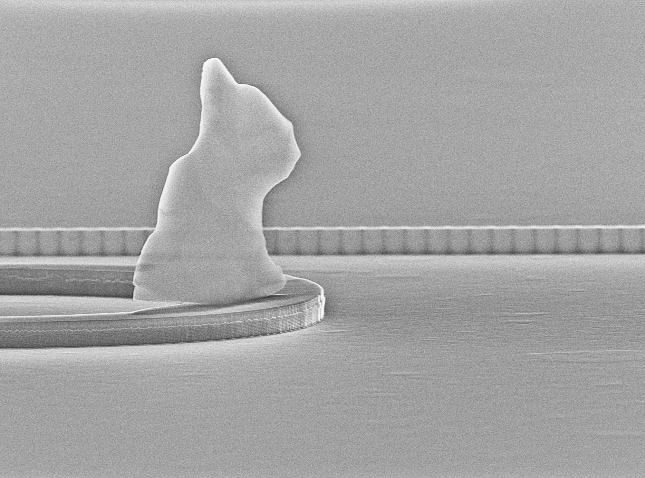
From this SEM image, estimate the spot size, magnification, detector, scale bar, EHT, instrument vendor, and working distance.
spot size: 3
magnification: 8.418 K X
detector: SE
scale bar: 2000 nm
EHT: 30 kV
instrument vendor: FEI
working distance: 15.7 mm